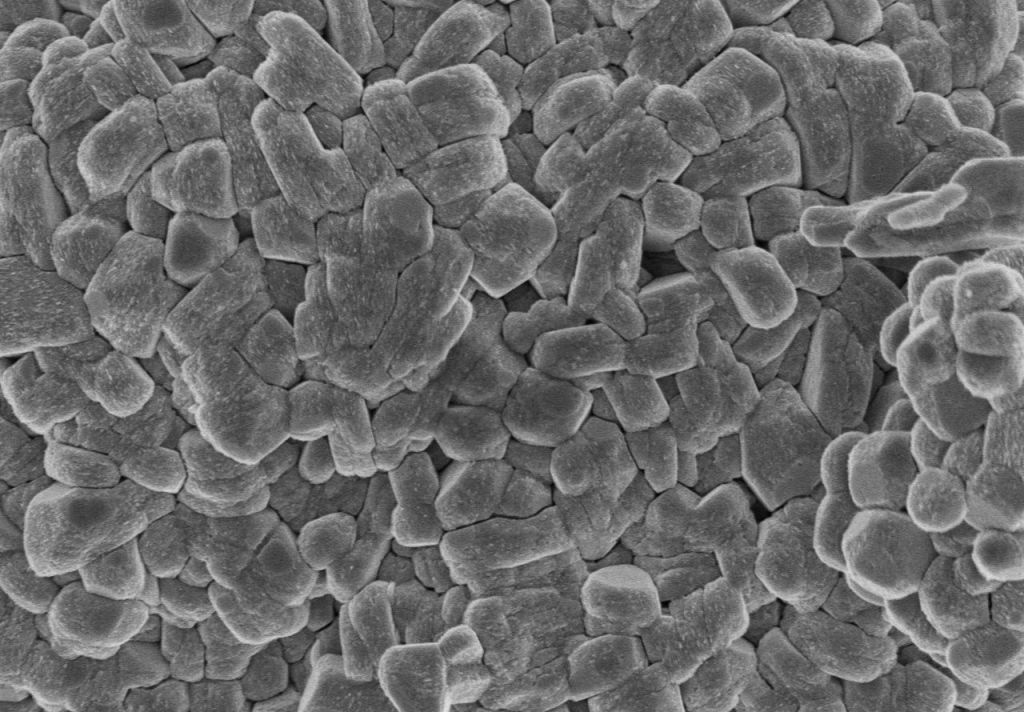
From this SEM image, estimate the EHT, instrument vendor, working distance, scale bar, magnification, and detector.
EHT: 3 kV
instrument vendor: Hitachi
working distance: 8.5 mm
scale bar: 2000 nm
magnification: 20 K X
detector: SE(M)